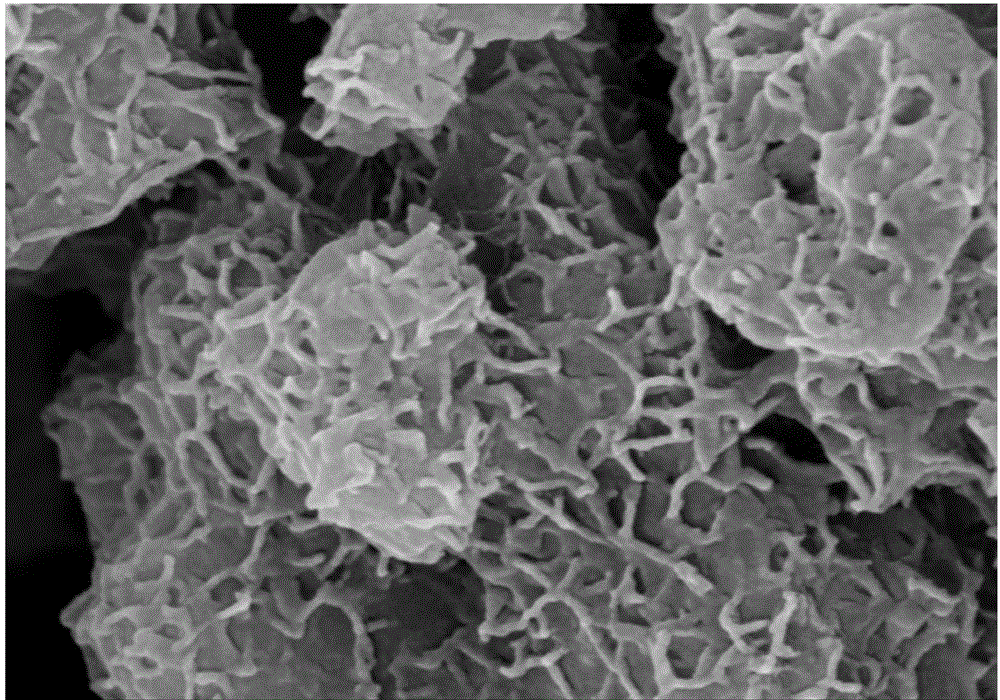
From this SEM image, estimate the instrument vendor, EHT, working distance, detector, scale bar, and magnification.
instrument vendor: Hitachi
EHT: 15 kV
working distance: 8.2 mm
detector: SE(M)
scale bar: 1000 nm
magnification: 50 K X